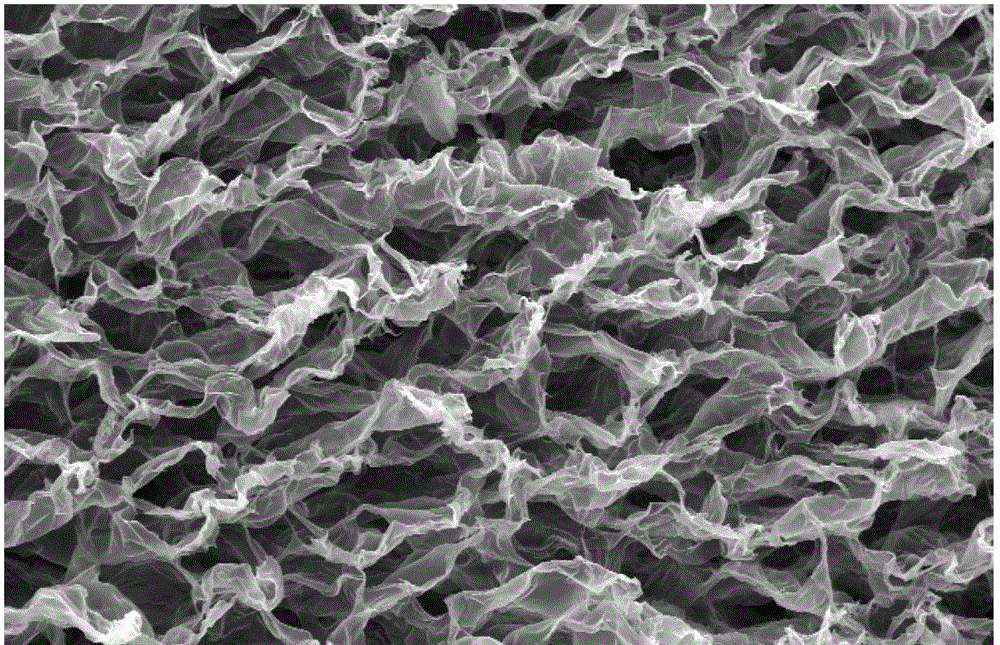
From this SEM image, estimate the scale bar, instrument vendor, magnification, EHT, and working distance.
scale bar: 100000 nm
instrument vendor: FEI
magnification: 0.5 K X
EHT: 20 kV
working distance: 9.9 mm